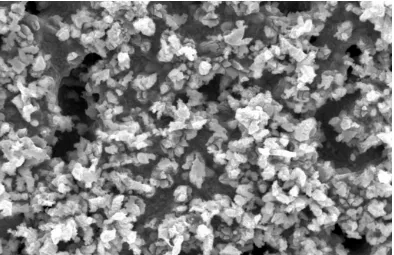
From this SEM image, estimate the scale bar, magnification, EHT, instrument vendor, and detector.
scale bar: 3000 nm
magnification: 3 K X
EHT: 20 kV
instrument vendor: Zeiss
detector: SE1